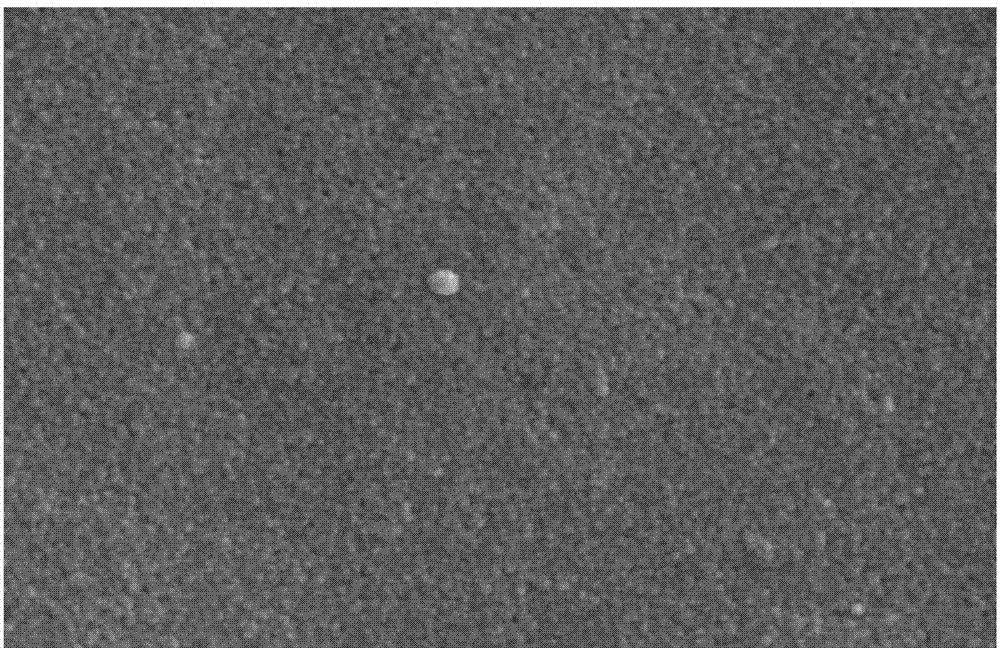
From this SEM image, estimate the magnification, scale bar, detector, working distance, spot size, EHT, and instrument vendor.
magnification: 100 K X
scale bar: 500 nm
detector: SE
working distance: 5.8 mm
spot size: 4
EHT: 25 kV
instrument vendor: FEI